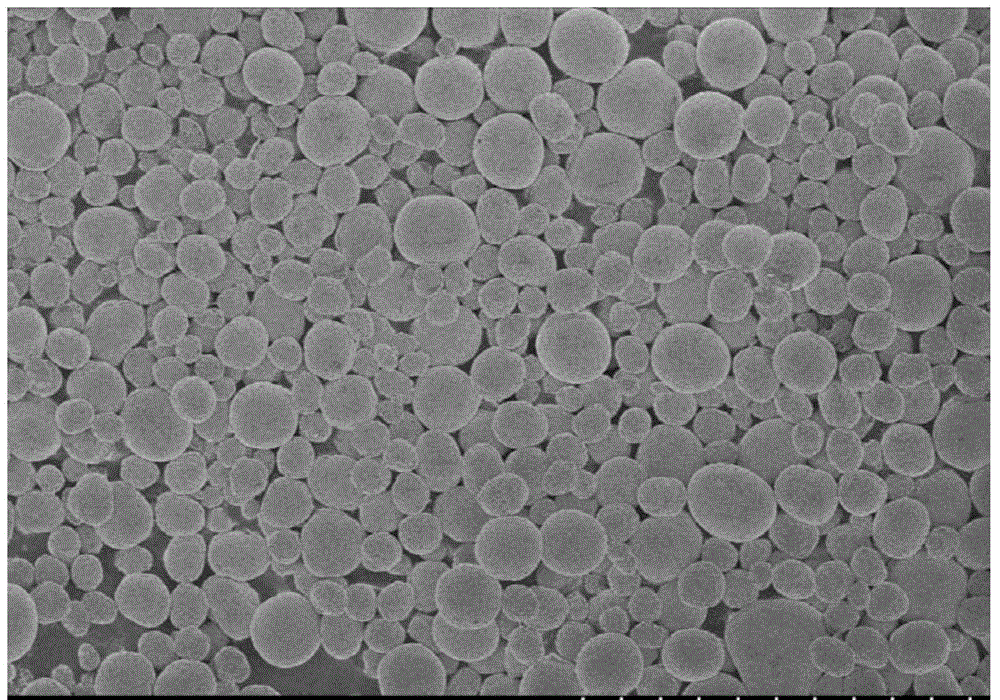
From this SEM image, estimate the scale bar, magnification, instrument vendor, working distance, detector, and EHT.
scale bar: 100000 nm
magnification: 0.5 K X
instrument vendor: Hitachi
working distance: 8 mm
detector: SE(M)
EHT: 3 kV